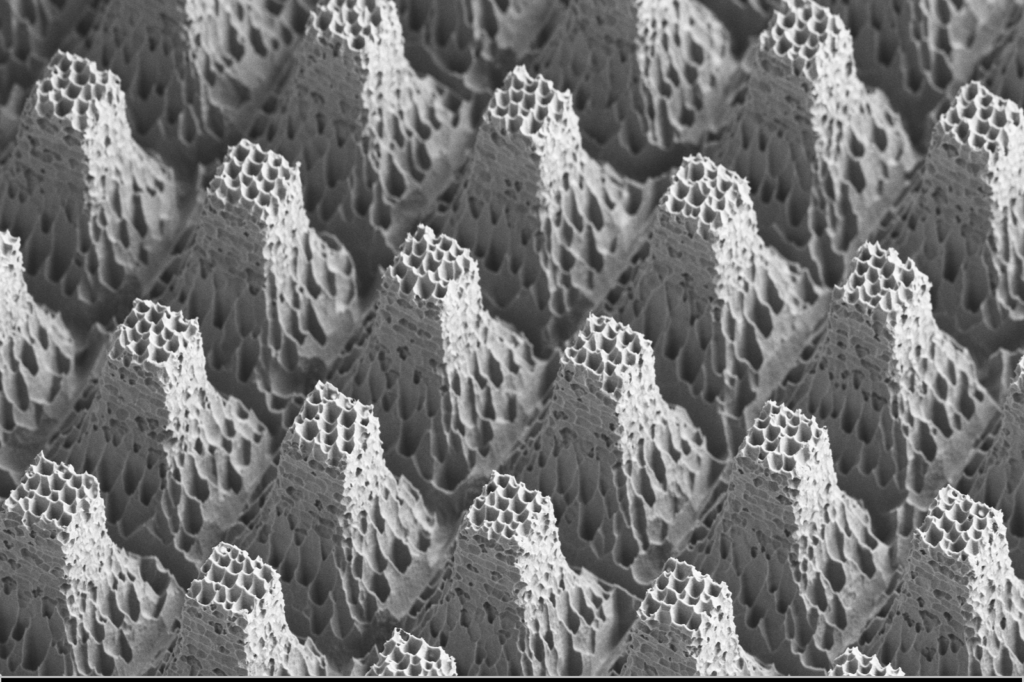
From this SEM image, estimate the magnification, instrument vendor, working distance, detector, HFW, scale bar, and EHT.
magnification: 4.06 K X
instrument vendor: Zeiss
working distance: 7.7 mm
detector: SE2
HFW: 74.26 µm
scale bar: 10000 nm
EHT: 3 kV